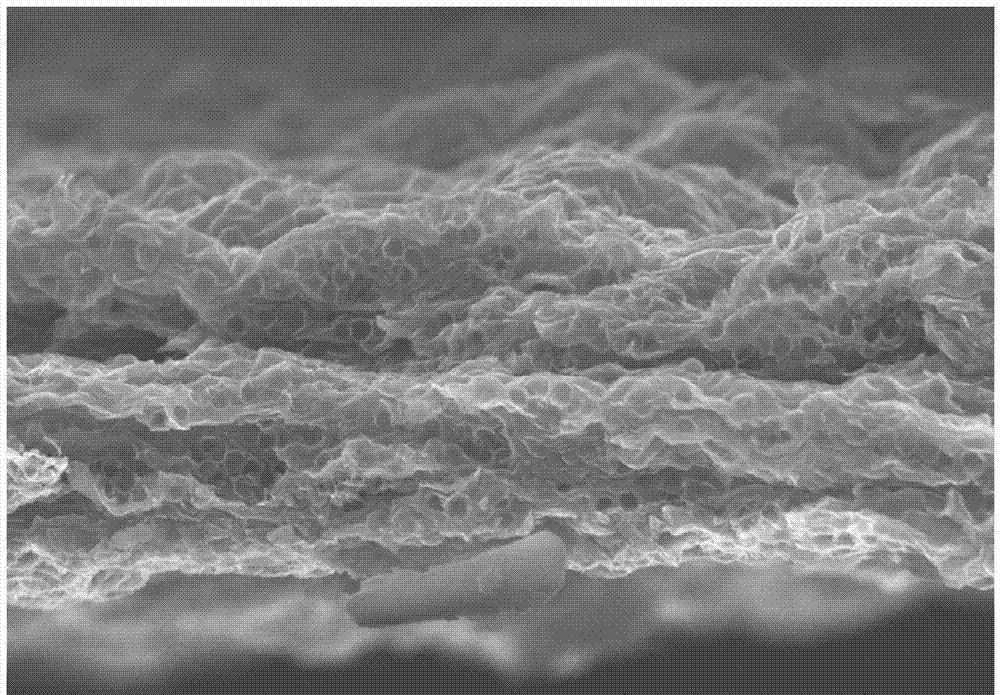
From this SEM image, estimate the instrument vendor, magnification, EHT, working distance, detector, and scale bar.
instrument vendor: Hitachi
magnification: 9 K X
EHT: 10 kV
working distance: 10 mm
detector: SE(U)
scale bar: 5000 nm